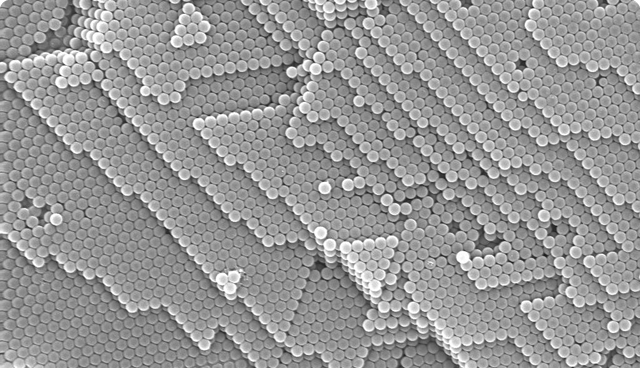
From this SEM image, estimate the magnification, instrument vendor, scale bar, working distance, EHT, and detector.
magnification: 10 K X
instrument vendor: Zeiss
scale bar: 1000 nm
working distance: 5 mm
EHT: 10 kV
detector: InLens